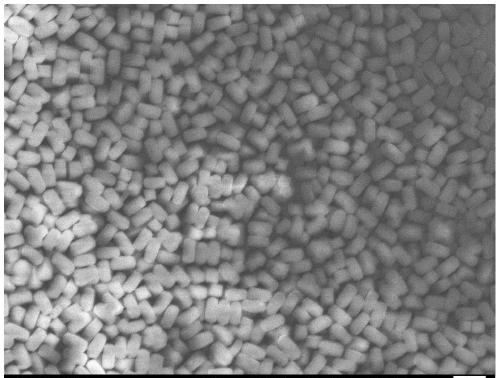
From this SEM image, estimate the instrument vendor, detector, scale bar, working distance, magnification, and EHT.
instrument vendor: JEOL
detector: SEI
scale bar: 1000 nm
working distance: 8 mm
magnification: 8 K X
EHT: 5 kV